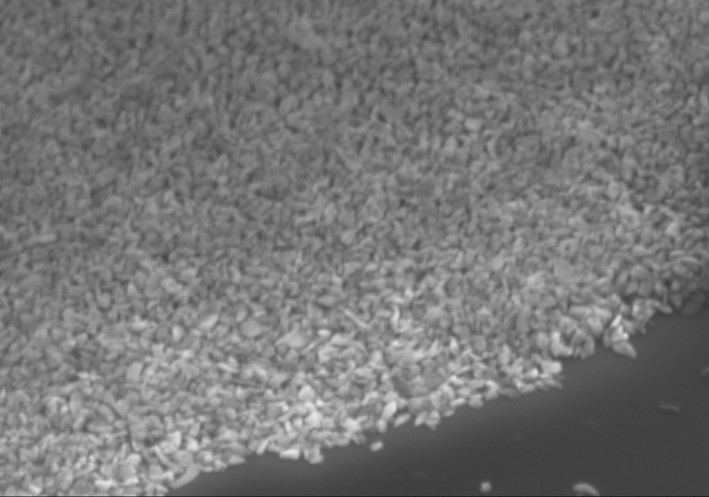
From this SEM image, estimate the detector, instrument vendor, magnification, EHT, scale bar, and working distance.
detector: SE(M)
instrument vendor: Hitachi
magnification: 100 K X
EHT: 3 kV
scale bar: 500 nm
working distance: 3.7 mm